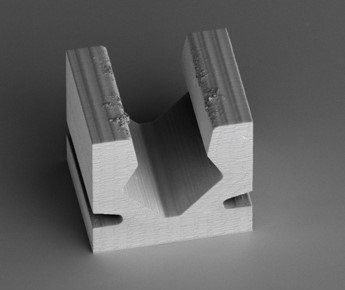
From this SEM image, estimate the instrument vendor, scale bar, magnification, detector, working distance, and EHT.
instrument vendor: FEI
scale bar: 100000 nm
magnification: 0.2 K X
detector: ETD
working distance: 8.4 mm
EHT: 2 kV